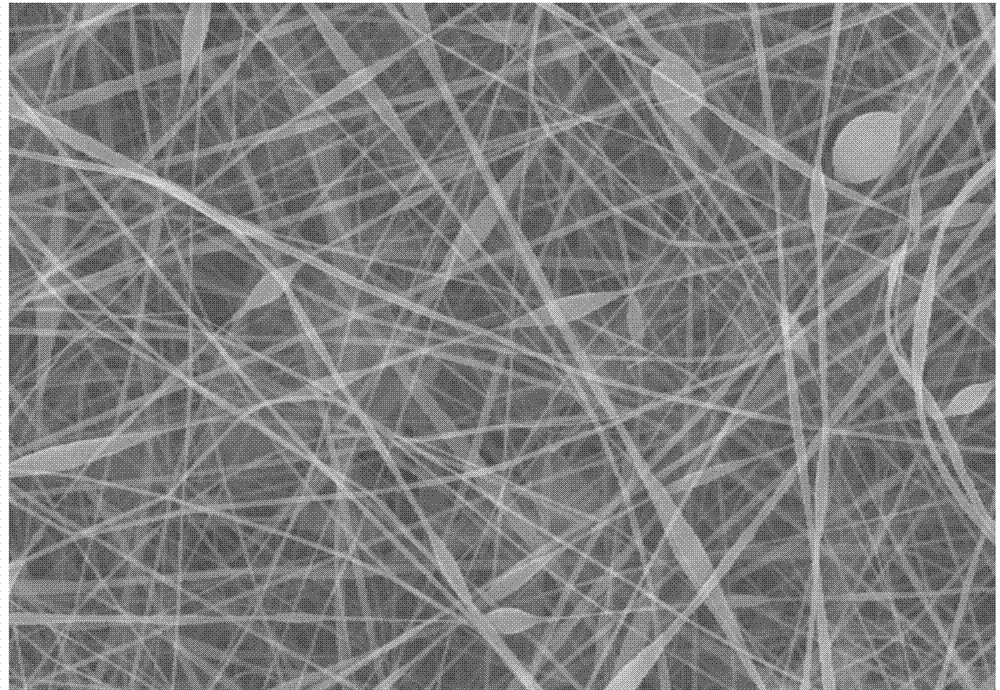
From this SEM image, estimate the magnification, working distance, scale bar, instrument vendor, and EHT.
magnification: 5 K X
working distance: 12.2 mm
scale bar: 10000 nm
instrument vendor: Hitachi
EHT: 20 kV